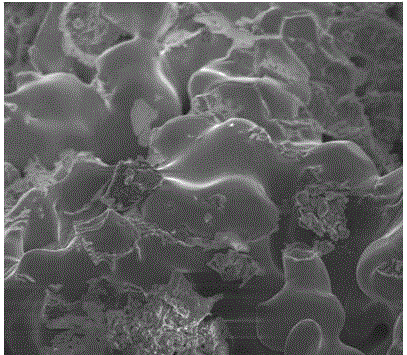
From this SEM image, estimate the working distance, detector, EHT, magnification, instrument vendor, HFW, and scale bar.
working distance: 5.5 mm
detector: TLD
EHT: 10 kV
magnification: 5 K X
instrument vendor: FEI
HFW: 48 µm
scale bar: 10000 nm